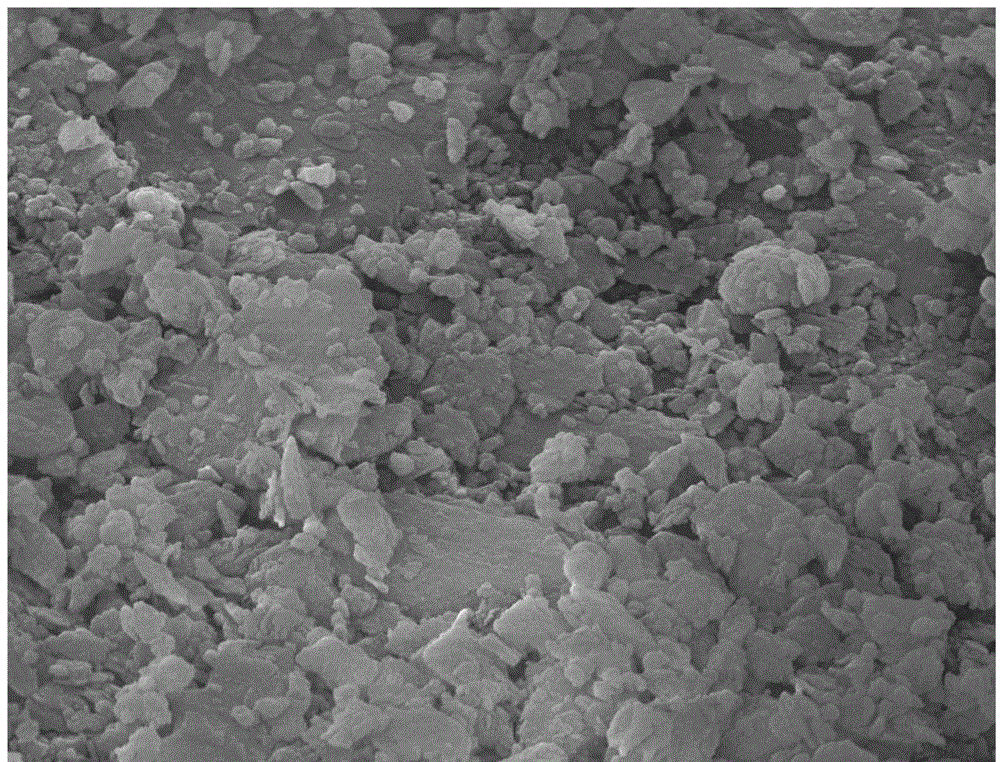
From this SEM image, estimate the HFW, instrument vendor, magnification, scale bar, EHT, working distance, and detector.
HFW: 9.01 µm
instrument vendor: FEI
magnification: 30 K X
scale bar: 2000 nm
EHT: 20 kV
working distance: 9.9 mm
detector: ETD